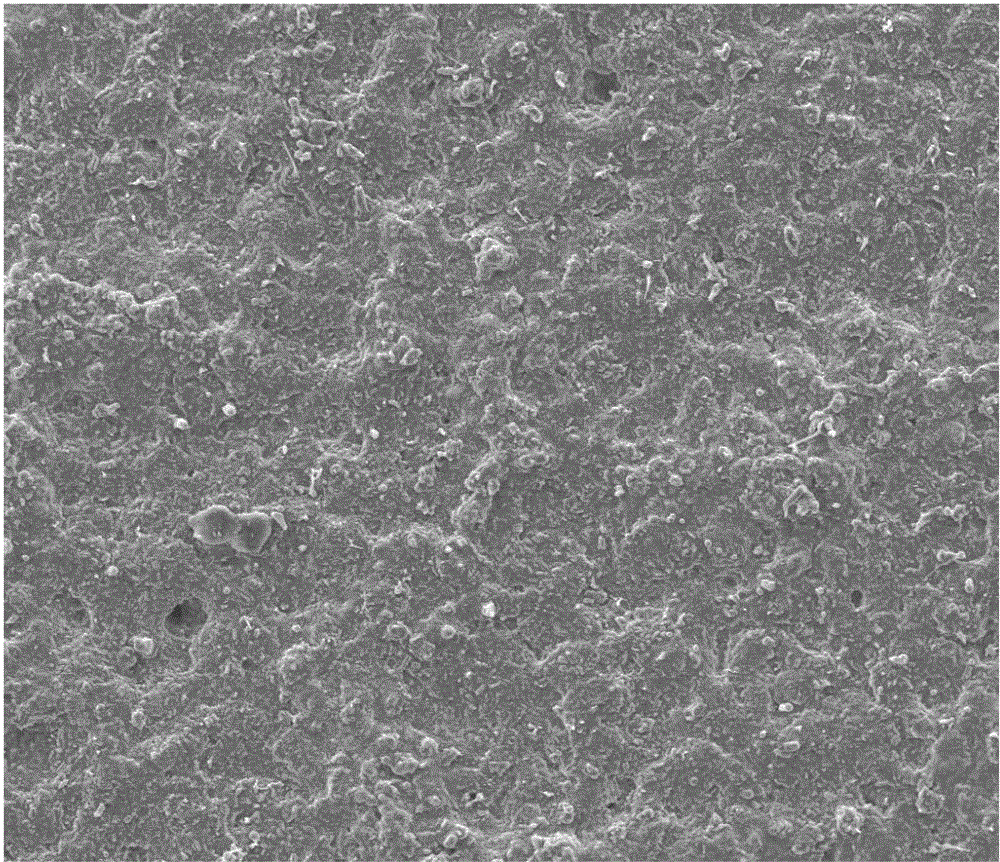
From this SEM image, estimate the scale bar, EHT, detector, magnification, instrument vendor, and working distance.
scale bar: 200000 nm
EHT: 20 kV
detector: ETD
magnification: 0.5 K X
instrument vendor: FEI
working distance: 10.3 mm